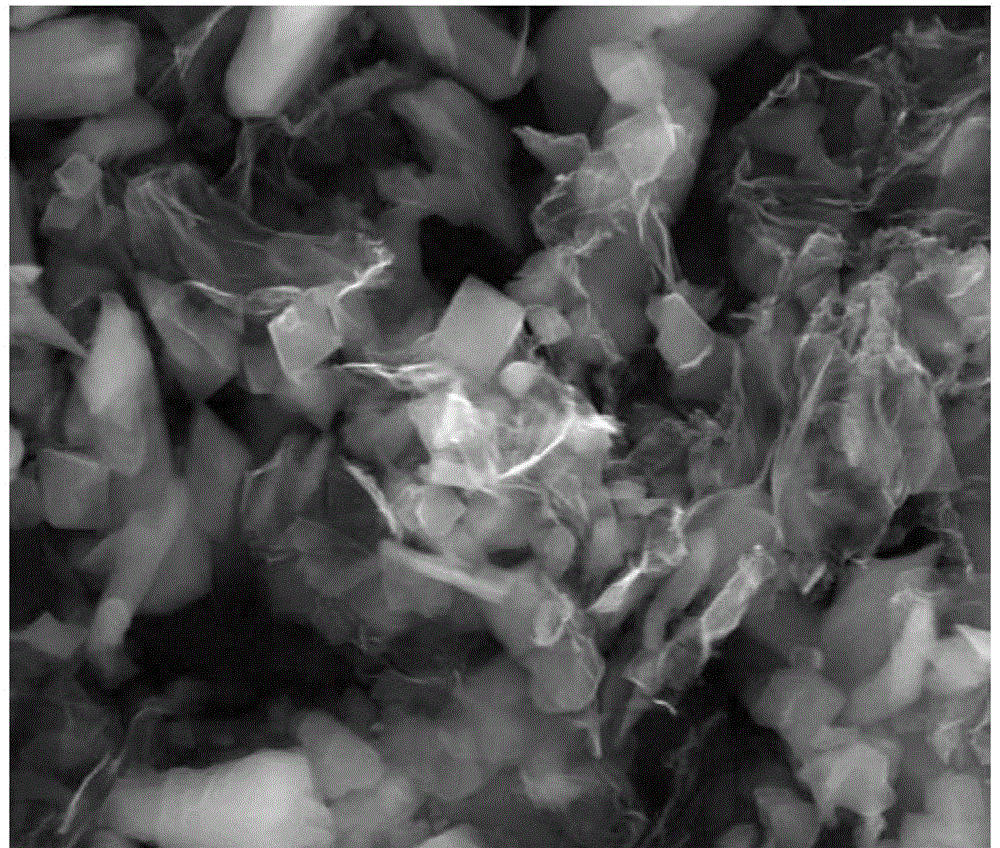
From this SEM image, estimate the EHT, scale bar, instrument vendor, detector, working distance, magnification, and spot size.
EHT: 20 kV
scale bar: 10000 nm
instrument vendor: FEI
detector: ETD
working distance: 10.7 mm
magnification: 10 K X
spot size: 4